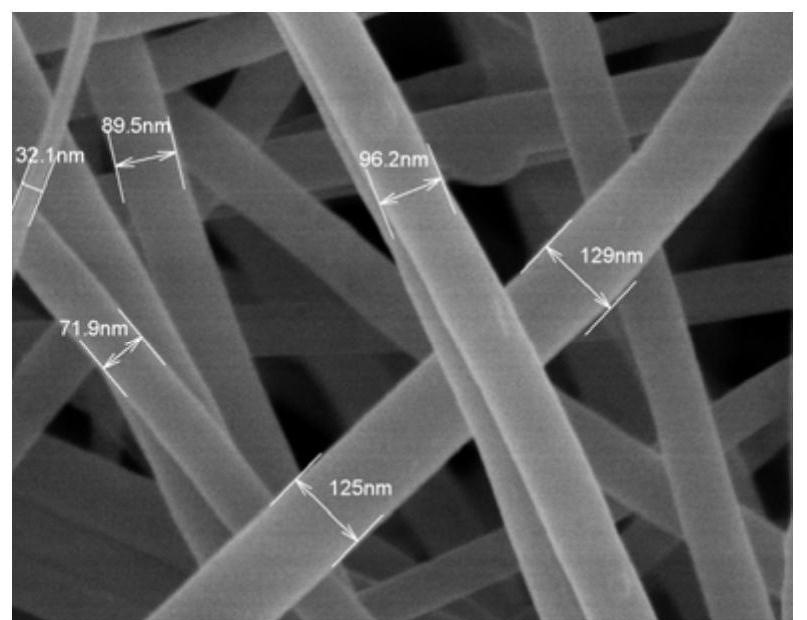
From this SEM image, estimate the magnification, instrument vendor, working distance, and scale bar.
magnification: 100 K X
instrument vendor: Hitachi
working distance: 7.4 mm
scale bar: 500 nm